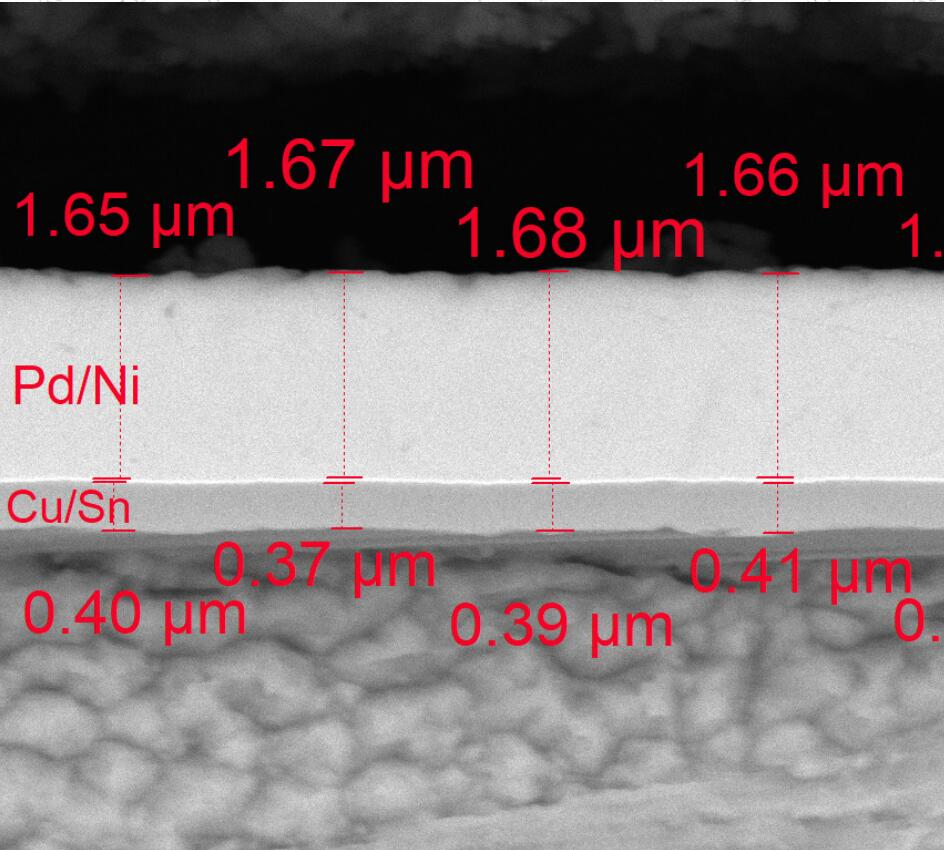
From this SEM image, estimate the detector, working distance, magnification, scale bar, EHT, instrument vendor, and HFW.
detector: LE-BSE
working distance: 14.9 mm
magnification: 30 K X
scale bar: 2000 nm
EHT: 15 kV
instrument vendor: Tescan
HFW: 9.23 µm